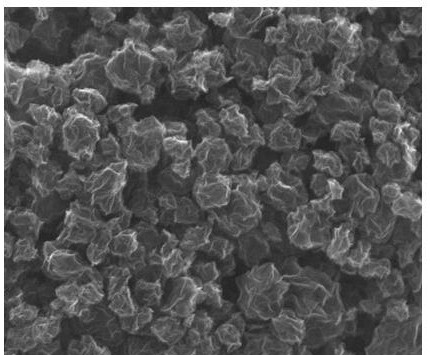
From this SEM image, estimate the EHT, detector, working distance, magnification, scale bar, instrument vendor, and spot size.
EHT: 20 kV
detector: ETD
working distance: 8.7 mm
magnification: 50 K X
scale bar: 2000 nm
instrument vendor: FEI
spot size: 3